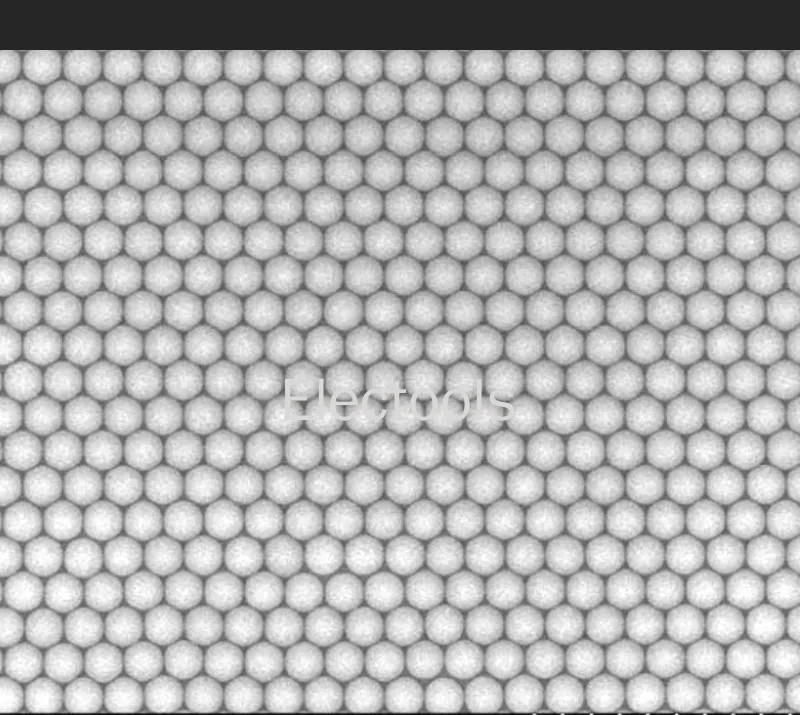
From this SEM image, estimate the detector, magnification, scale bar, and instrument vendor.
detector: SE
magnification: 3.5 K X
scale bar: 10000 nm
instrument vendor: Hitachi